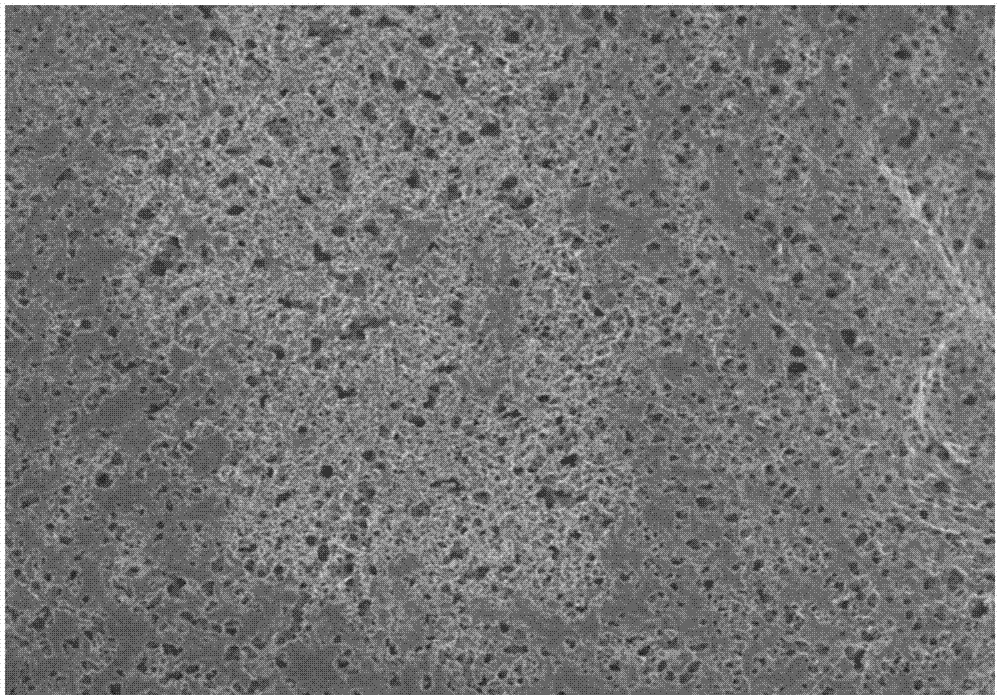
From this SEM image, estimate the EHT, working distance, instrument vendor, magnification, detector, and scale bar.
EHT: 3 kV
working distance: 9.7 mm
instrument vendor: Hitachi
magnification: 1 K X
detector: SE(M)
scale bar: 50000 nm